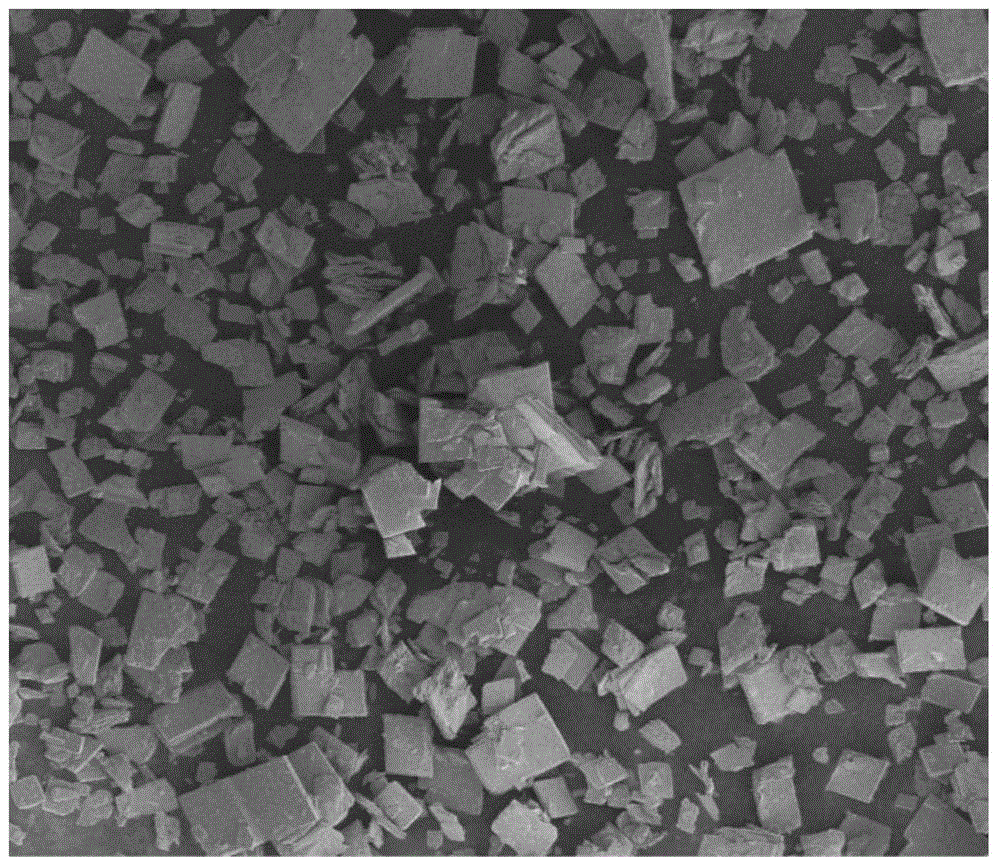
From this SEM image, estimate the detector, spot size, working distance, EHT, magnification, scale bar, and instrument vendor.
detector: ETD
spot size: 2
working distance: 3 mm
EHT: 3 kV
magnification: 0.2 K X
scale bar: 200000 nm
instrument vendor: FEI